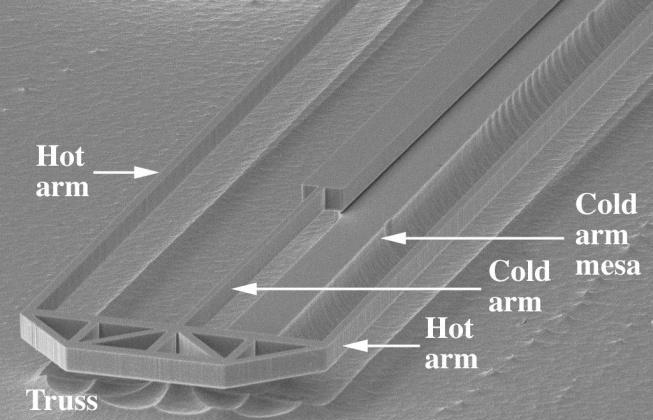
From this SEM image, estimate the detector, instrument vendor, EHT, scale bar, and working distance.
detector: SE1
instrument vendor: Zeiss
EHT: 20 kV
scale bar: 100000 nm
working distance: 48 mm